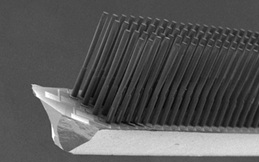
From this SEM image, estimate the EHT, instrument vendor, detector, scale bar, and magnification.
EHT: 2 kV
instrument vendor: JEOL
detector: SEI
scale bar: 500000 nm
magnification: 0.05 K X